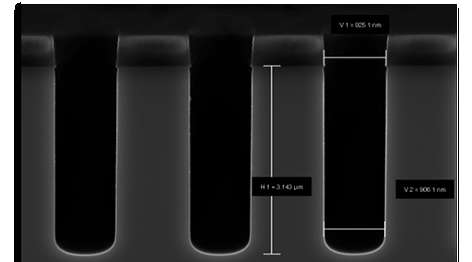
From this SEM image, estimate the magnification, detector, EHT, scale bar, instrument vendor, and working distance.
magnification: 17.67 K X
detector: InLens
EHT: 5 kV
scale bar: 1000 nm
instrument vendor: Zeiss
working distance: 2 mm